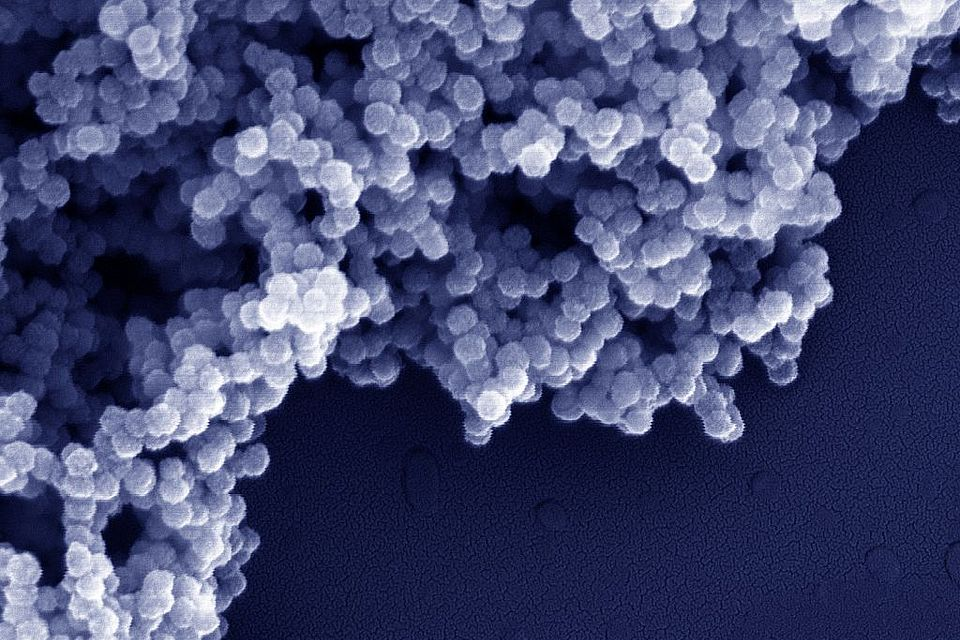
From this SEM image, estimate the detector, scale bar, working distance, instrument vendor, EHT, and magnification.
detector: InLens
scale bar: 200 nm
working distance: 3 mm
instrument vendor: Zeiss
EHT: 10 kV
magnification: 150 K X